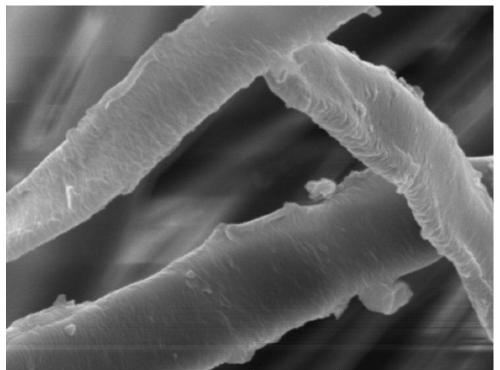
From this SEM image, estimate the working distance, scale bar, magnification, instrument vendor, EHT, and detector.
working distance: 12.55 mm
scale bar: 5000 nm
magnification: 10 K X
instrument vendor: Tescan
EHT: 10 kV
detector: SE